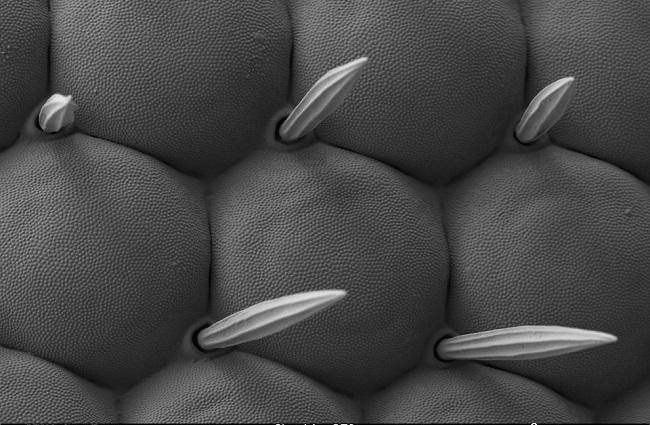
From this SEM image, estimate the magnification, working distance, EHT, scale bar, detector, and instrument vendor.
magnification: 3 K X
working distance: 4.2 mm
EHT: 1 kV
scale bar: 2000 nm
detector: SE2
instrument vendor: Zeiss